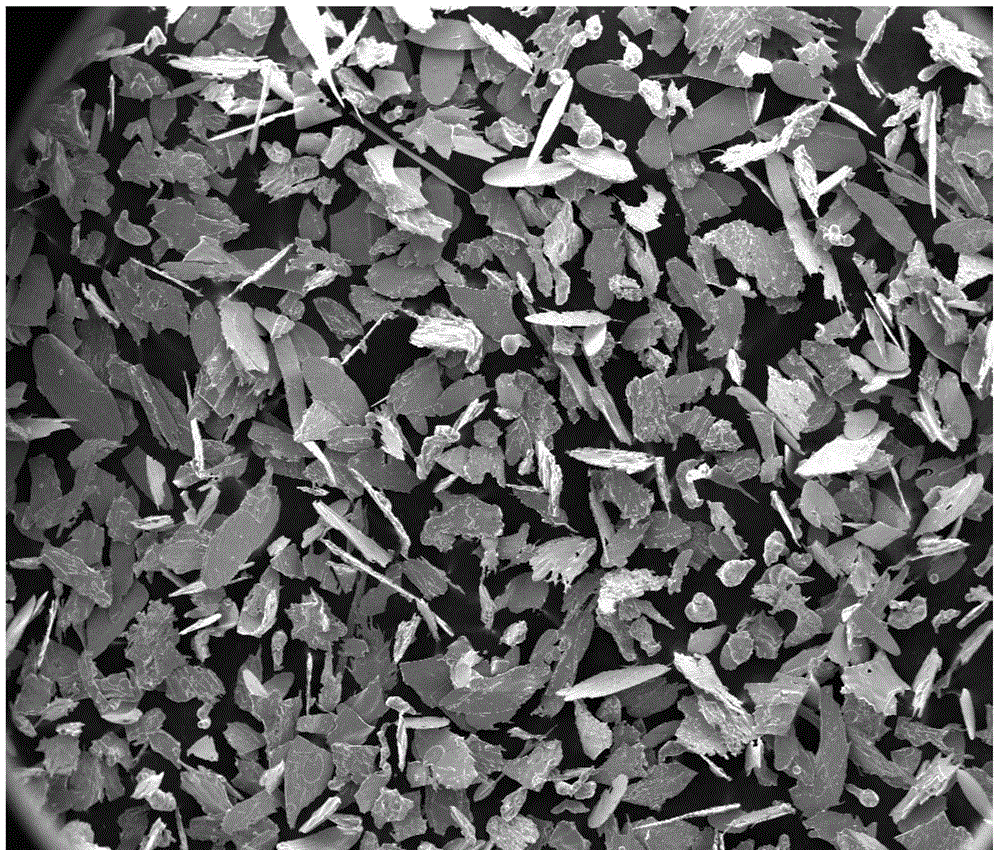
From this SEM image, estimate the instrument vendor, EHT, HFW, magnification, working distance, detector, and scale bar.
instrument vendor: FEI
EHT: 10 kV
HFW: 2540 µm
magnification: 0.05 K X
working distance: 10.2 mm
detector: ETD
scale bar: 1000000 nm